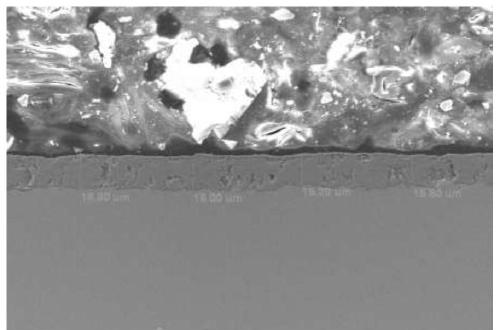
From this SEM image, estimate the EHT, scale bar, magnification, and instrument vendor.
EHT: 15 kV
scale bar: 50000 nm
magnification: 0.5 K X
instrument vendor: JEOL